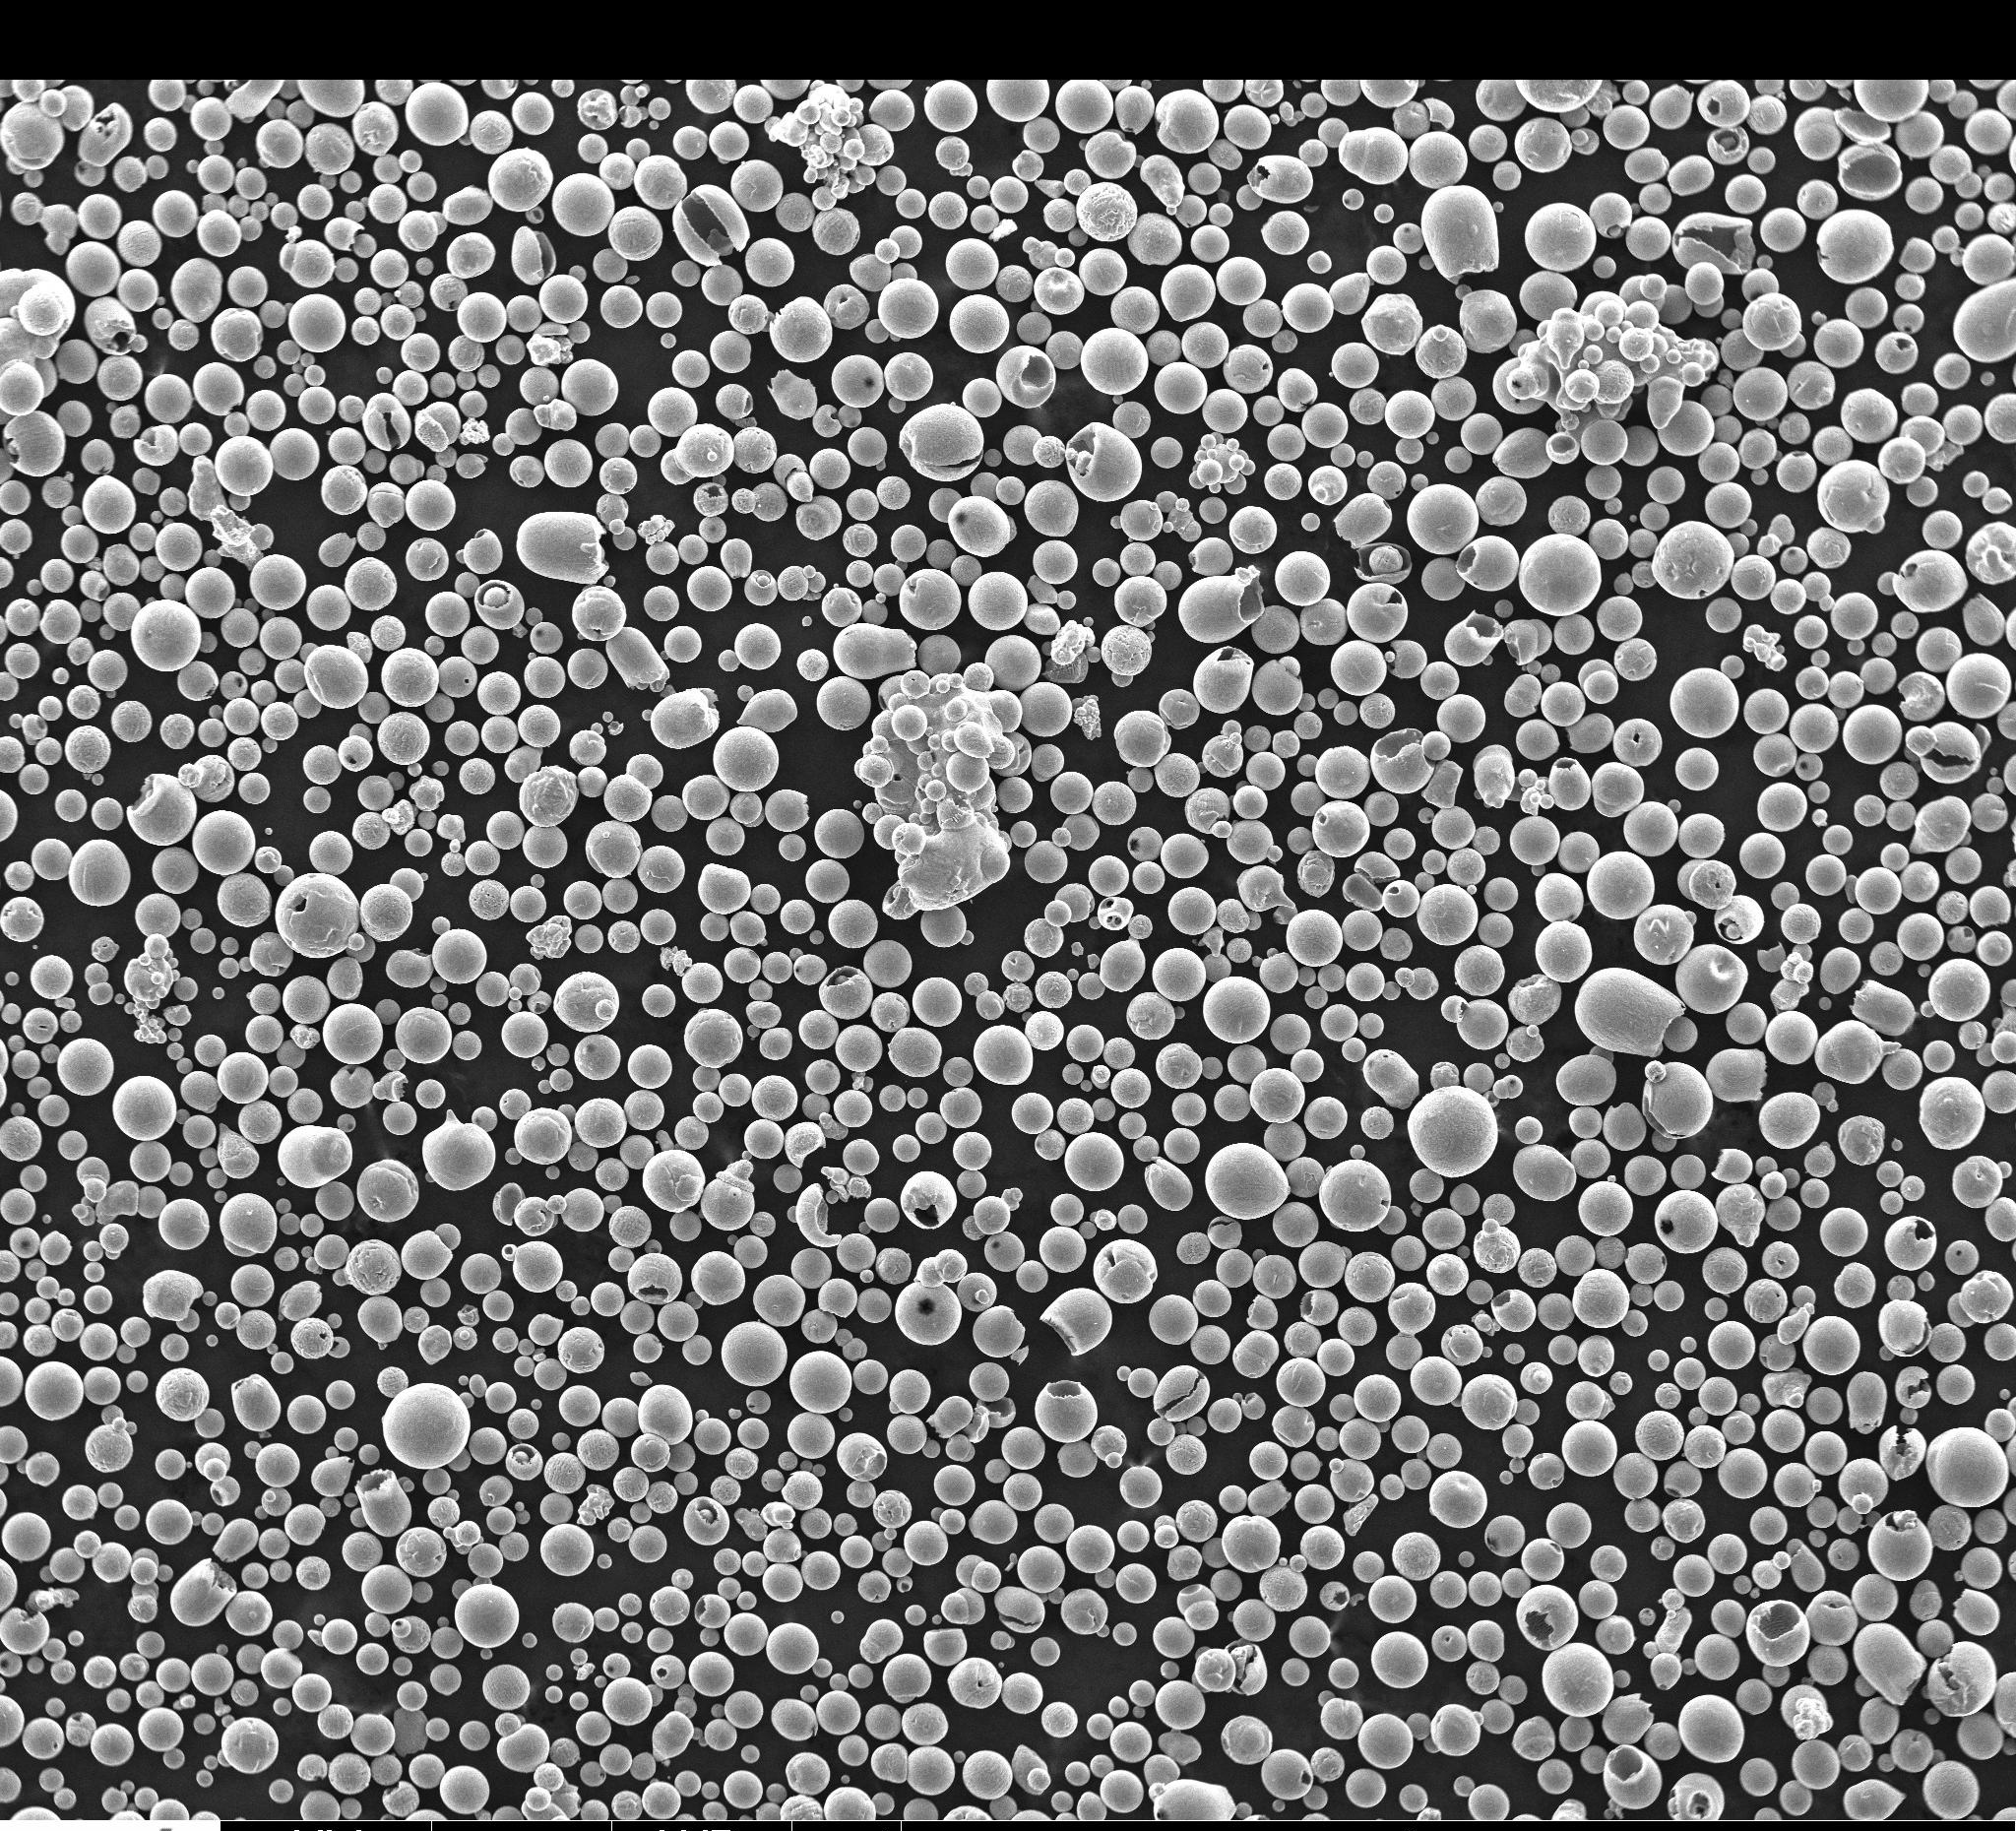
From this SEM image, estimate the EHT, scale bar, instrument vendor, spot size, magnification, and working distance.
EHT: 30 kV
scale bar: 1000000 nm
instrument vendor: FEI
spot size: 3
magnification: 0.046 K X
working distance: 9.7 mm